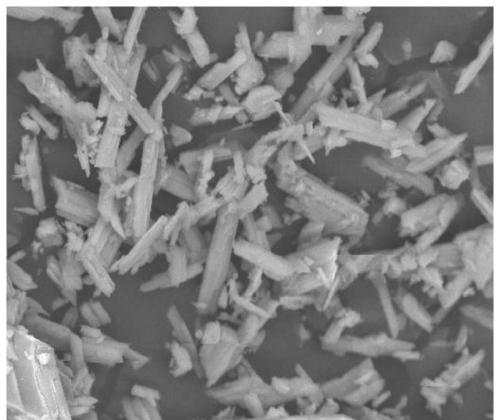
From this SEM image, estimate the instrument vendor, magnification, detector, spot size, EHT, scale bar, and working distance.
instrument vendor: FEI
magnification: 2 K X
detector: BSED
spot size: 4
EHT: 20 kV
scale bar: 30000 nm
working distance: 21.1 mm